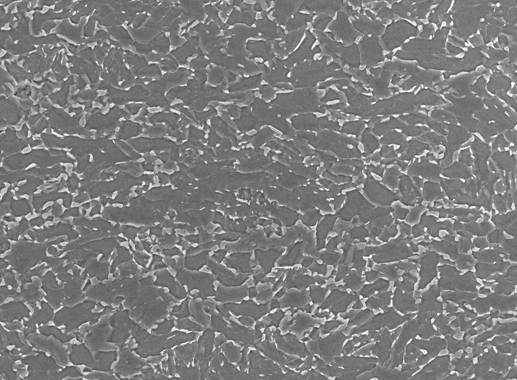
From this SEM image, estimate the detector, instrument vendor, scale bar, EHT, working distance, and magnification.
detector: SEI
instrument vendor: JEOL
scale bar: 10000 nm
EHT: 20 kV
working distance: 8.5 mm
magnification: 1 K X